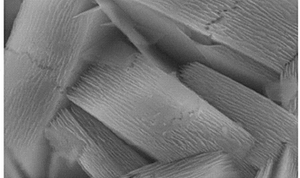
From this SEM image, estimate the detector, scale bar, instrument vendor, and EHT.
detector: InLens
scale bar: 200 nm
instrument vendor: Zeiss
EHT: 10 kV